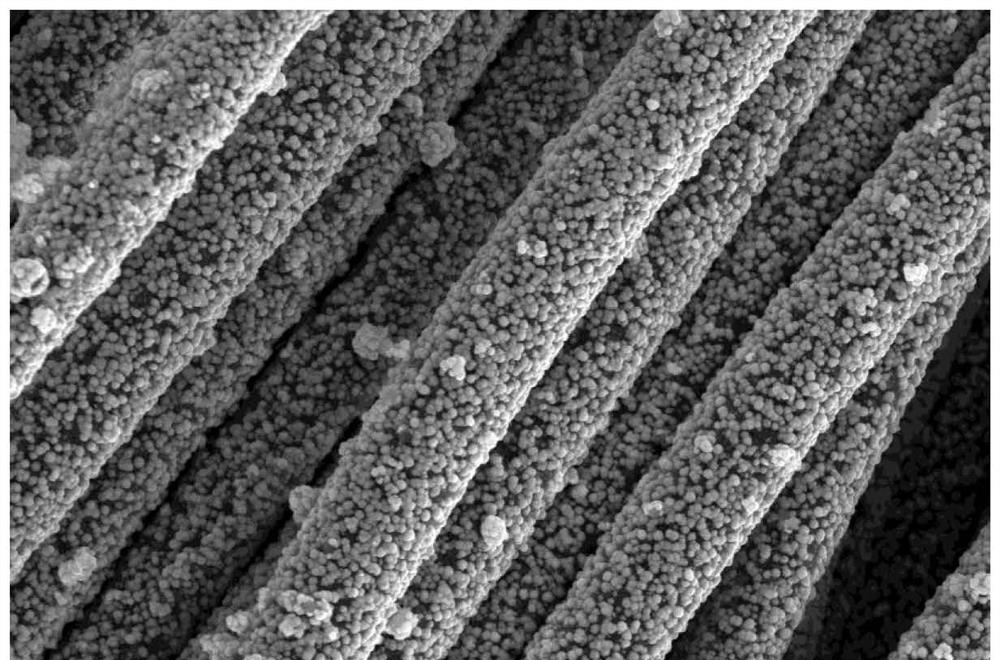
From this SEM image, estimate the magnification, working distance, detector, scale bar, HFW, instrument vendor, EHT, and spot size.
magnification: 5 K X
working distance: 10.5 mm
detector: ETD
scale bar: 20000 nm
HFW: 82.9 µm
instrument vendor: FEI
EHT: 15 kV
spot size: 10